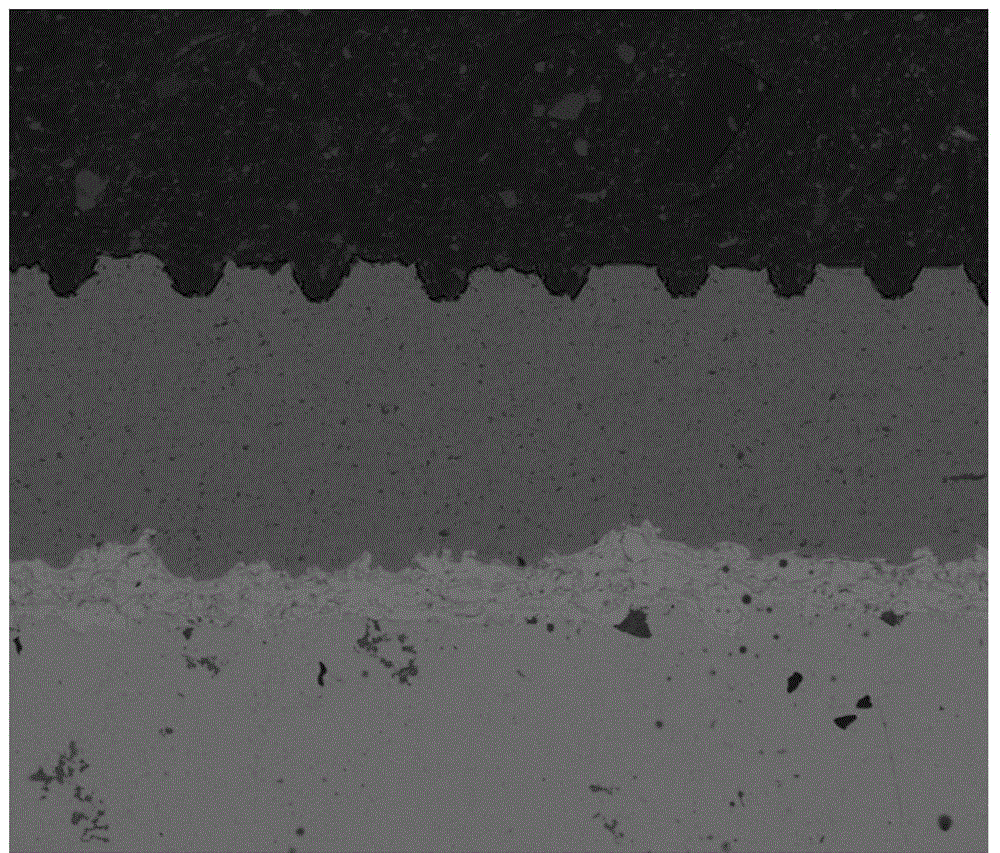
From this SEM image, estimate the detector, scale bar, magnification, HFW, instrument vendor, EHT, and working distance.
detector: vCD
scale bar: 500000 nm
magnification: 0.1 K X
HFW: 1270 µm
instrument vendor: FEI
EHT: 10 kV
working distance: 9.7 mm